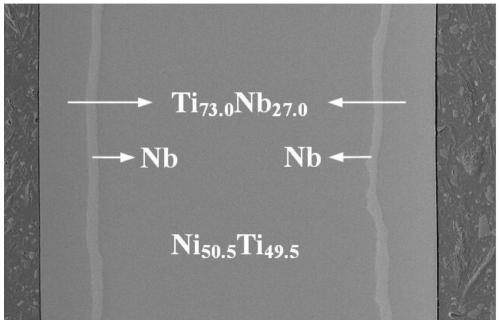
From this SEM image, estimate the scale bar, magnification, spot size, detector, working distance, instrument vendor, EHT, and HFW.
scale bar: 200000 nm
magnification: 0.3 K X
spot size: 3.5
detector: ETD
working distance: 5.6 mm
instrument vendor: FEI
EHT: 5 kV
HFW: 691 µm